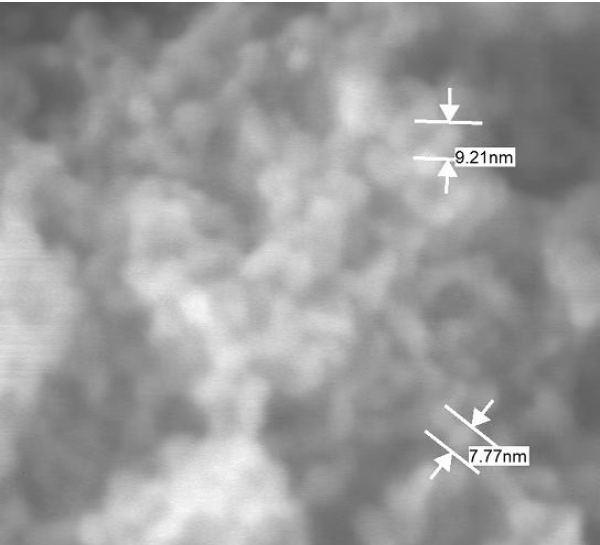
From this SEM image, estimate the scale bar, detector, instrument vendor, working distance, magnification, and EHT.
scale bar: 10 nm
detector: SEI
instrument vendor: JEOL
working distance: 2.9 mm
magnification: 550 K X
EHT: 5 kV